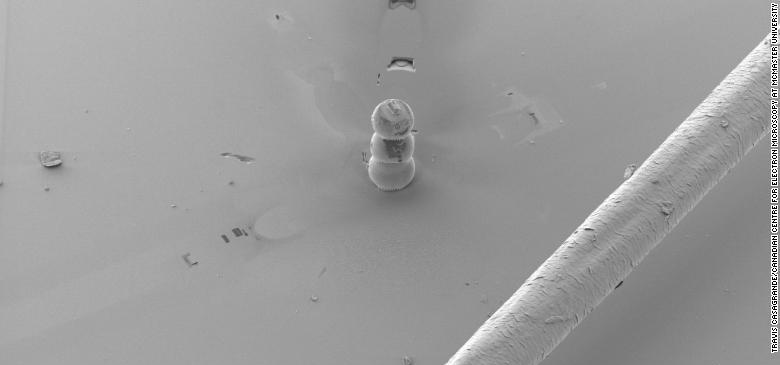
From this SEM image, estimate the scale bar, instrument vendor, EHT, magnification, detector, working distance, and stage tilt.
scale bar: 20000 nm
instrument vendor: Zeiss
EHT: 7 kV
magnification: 0.125 K X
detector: SESI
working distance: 6.8 mm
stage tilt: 40°